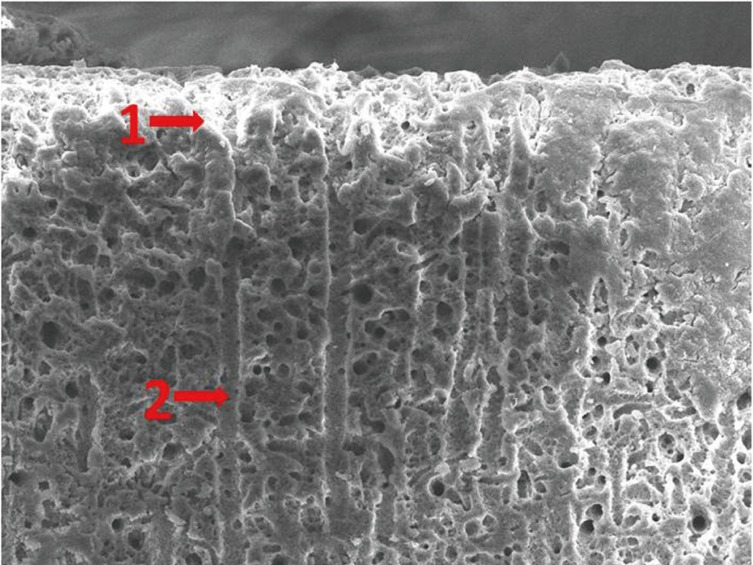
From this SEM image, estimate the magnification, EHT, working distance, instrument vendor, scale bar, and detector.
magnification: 1 K X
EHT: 10 kV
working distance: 10 mm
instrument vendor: JEOL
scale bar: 10000 nm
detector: SEI